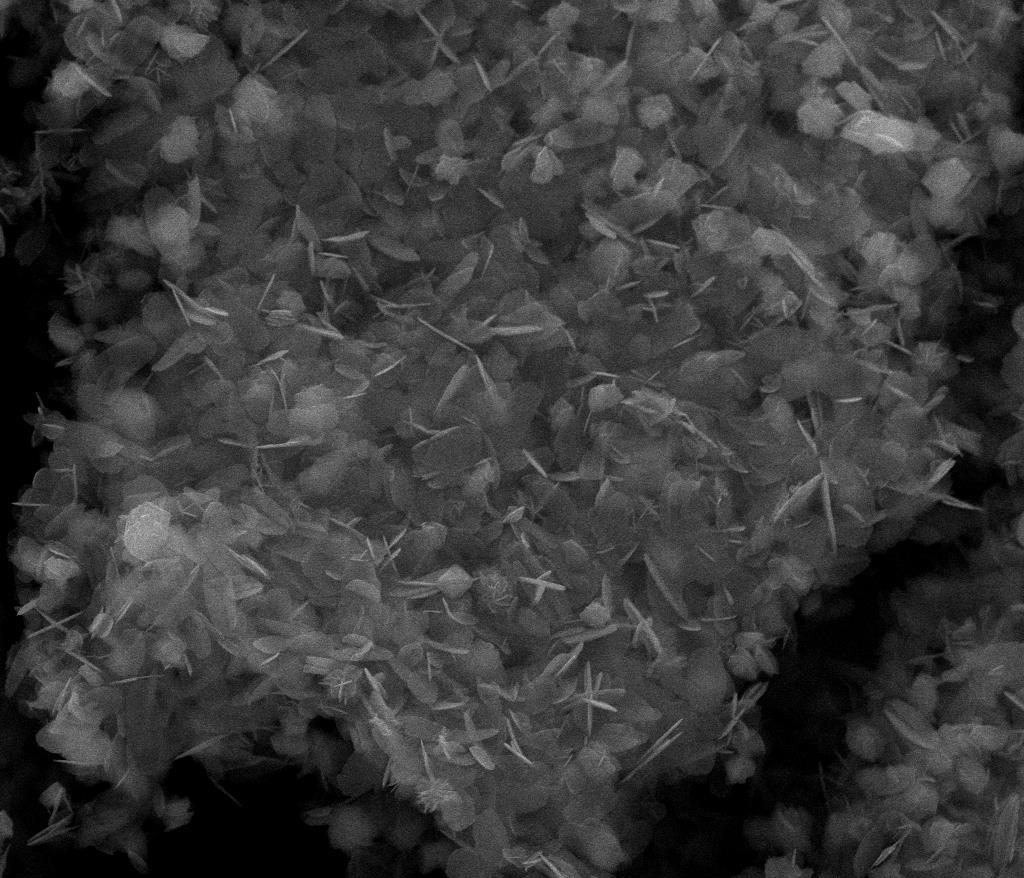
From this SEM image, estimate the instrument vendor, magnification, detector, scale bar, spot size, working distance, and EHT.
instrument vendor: FEI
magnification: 5 K X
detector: ETD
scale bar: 20000 nm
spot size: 3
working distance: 10.7 mm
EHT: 30 kV